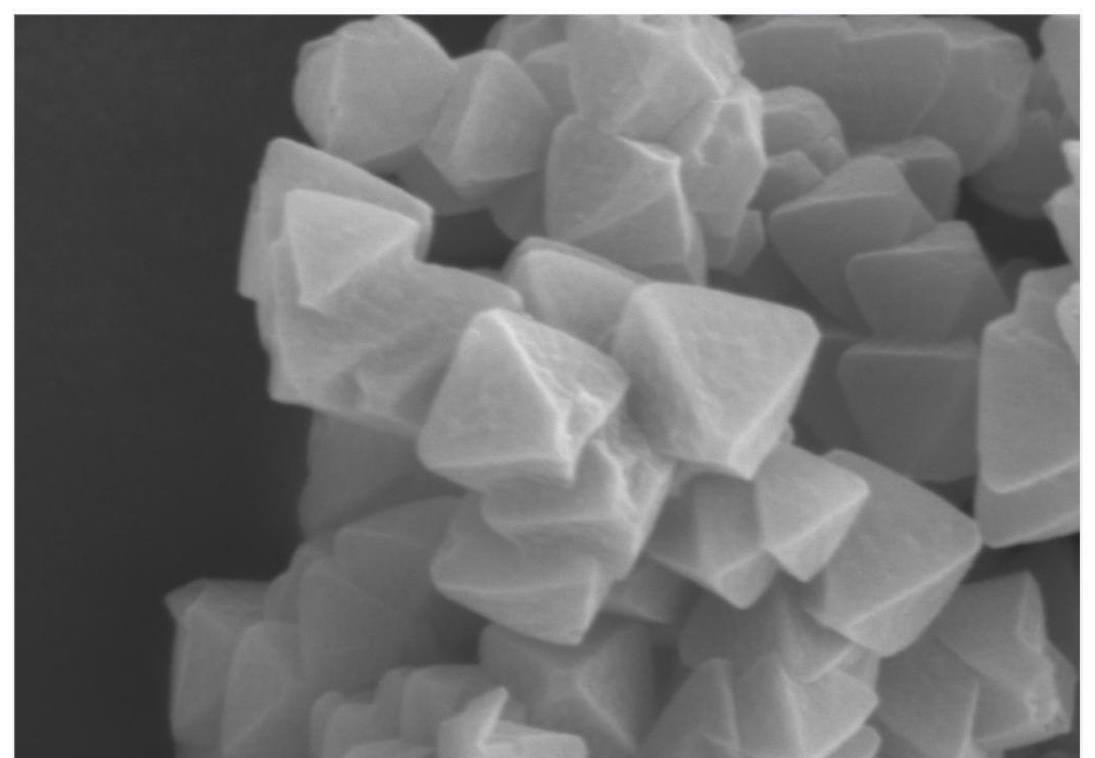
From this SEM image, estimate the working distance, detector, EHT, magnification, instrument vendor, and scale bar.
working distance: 6.9 mm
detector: SE(L)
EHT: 5 kV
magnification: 60 K X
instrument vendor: Hitachi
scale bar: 500 nm